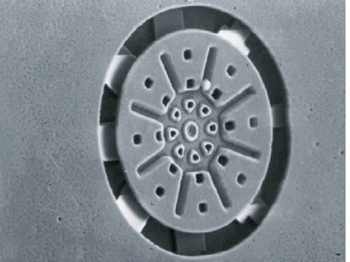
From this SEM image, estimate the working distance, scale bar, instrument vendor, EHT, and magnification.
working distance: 13 mm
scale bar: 10000 nm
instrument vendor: JEOL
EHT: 10 kV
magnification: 1.4 K X